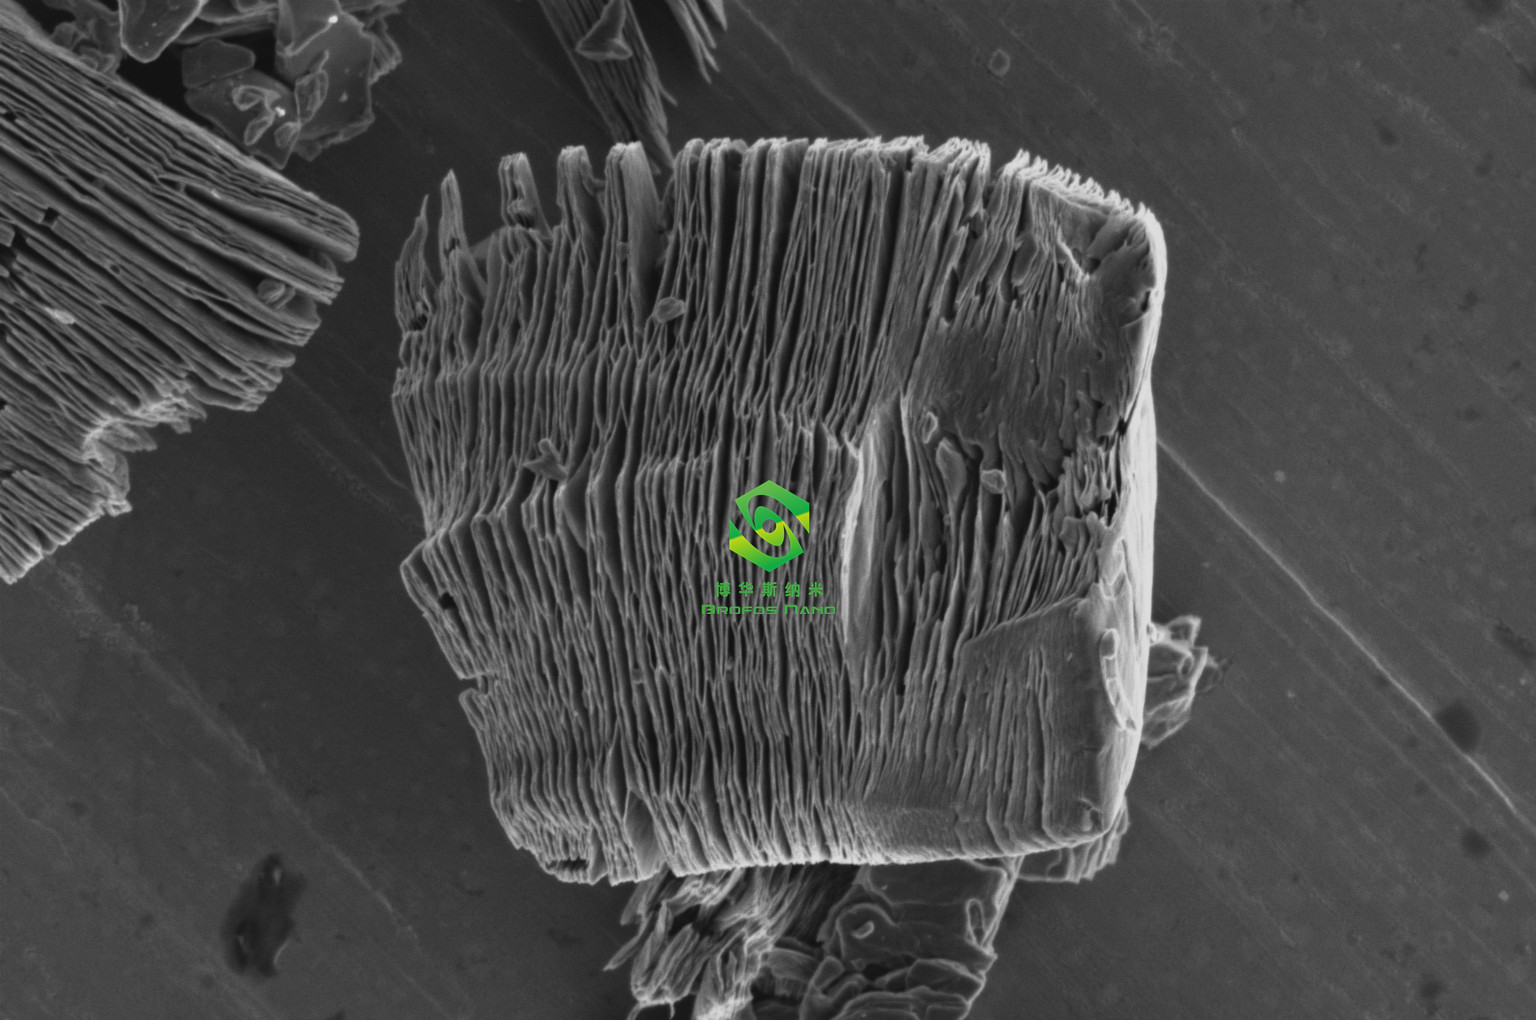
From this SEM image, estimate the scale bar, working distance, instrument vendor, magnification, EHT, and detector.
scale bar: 5000 nm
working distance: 9.9 mm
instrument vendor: Thermo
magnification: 10 K X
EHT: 2 kV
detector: T2